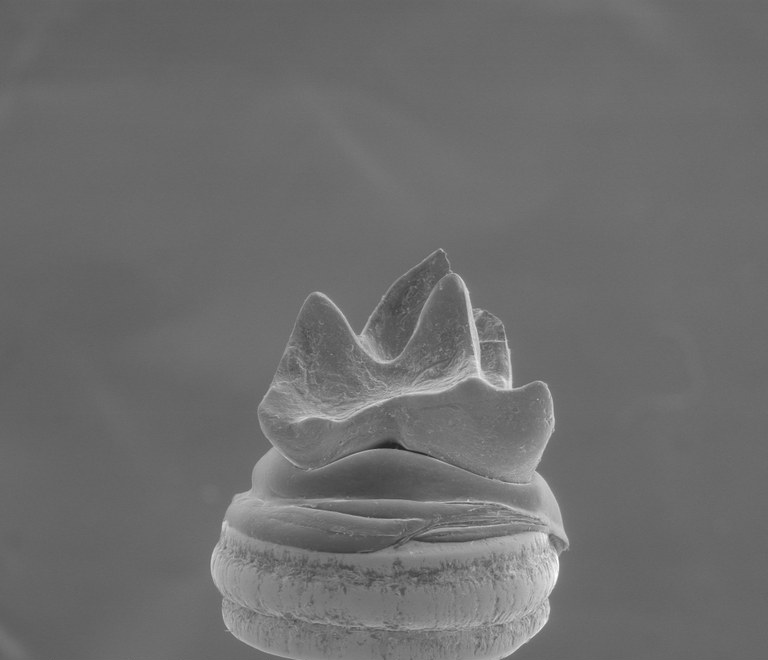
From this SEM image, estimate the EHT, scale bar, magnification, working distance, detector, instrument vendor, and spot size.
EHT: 20 kV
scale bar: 1000000 nm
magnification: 0.09 K X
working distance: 21.2 mm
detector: LFD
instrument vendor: FEI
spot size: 4